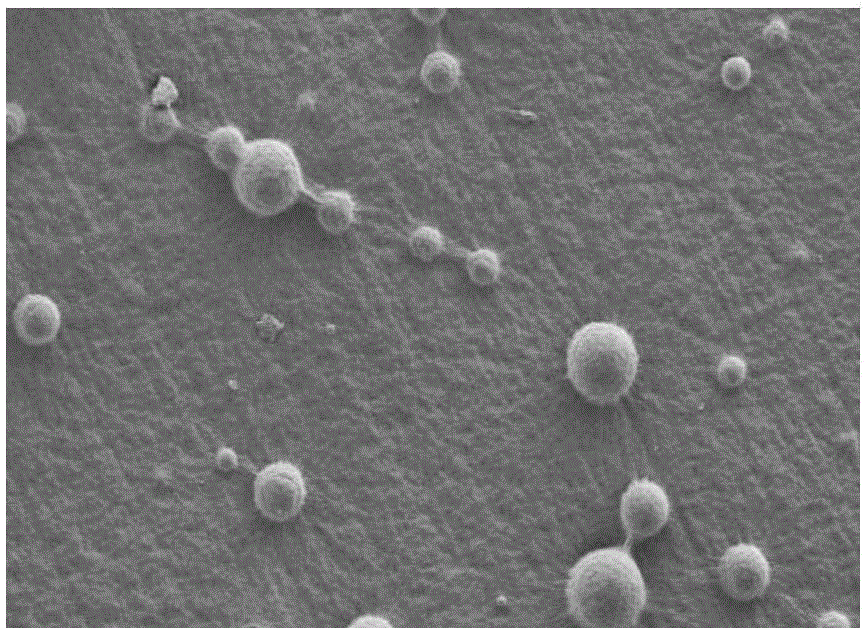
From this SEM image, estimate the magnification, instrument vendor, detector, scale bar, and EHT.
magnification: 0.11 K X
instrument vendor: Hitachi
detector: SE(M)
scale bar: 500000 nm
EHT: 10 kV